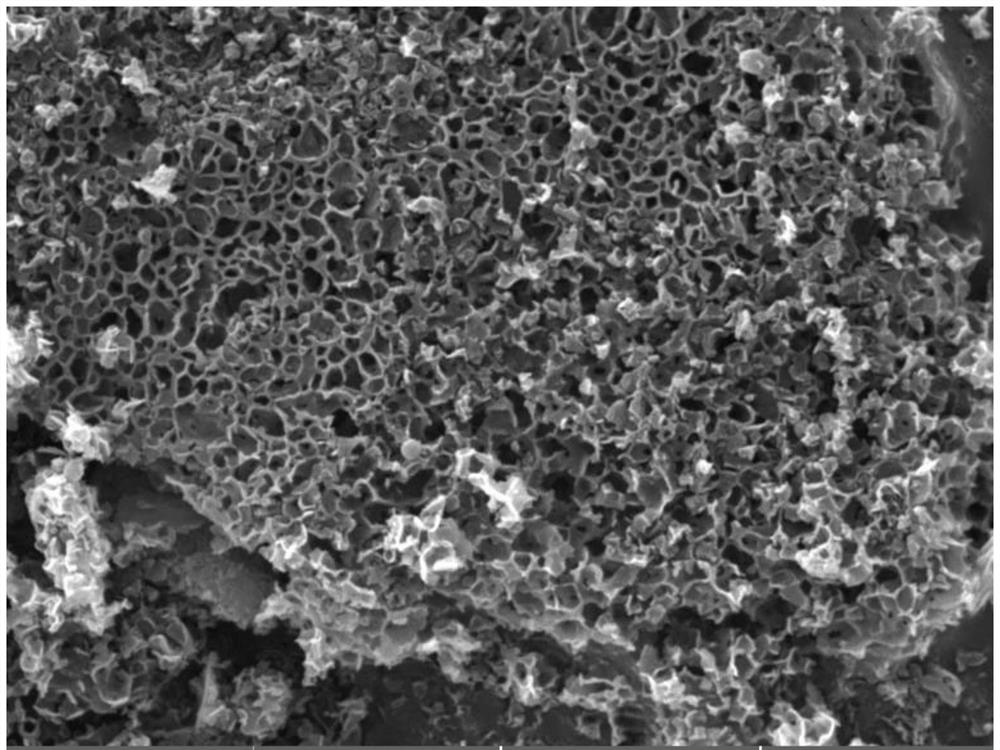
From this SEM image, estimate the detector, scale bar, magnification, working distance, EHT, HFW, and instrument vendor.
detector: SE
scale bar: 5000 nm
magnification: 10 K X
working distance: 8.77 mm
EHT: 20 kV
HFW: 19 µm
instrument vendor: Tescan